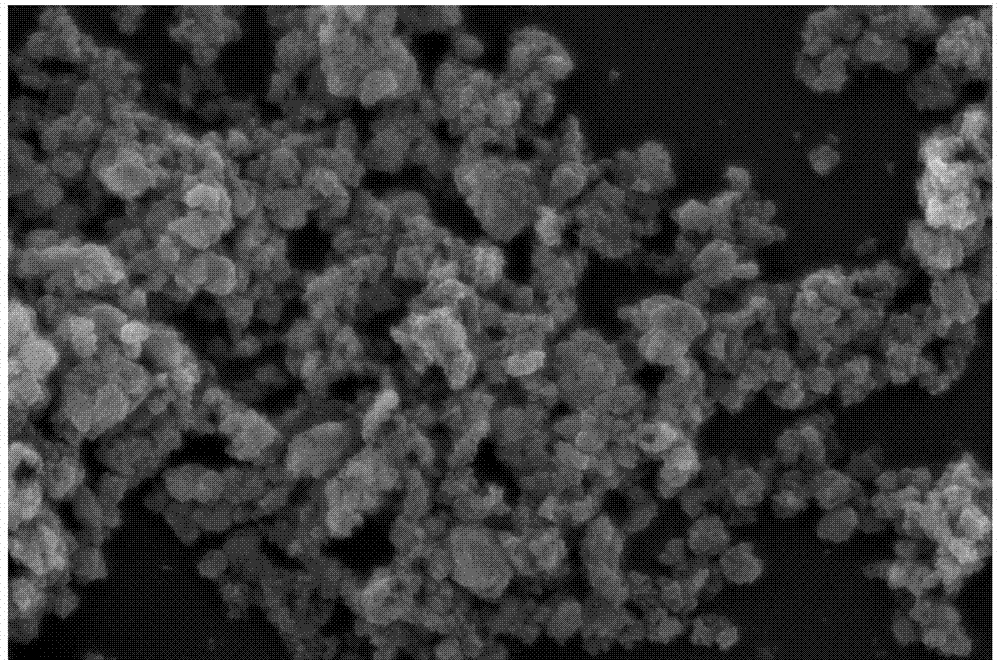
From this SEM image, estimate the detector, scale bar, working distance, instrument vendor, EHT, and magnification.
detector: SE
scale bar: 5000 nm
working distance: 7.8 mm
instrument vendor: Hitachi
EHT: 25 kV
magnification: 6 K X